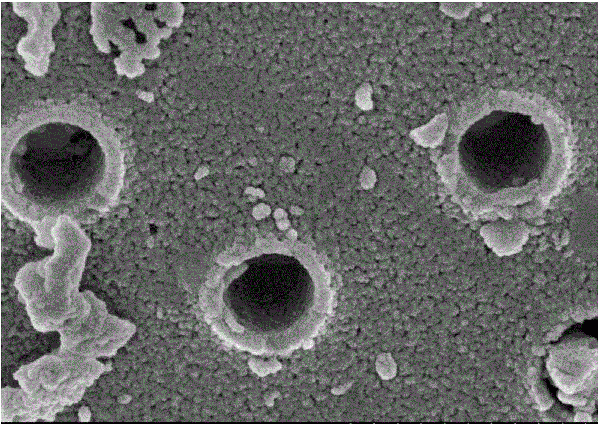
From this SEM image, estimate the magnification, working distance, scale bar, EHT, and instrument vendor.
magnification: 50 K X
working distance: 2.4 mm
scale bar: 1000 nm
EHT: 3 kV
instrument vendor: Hitachi